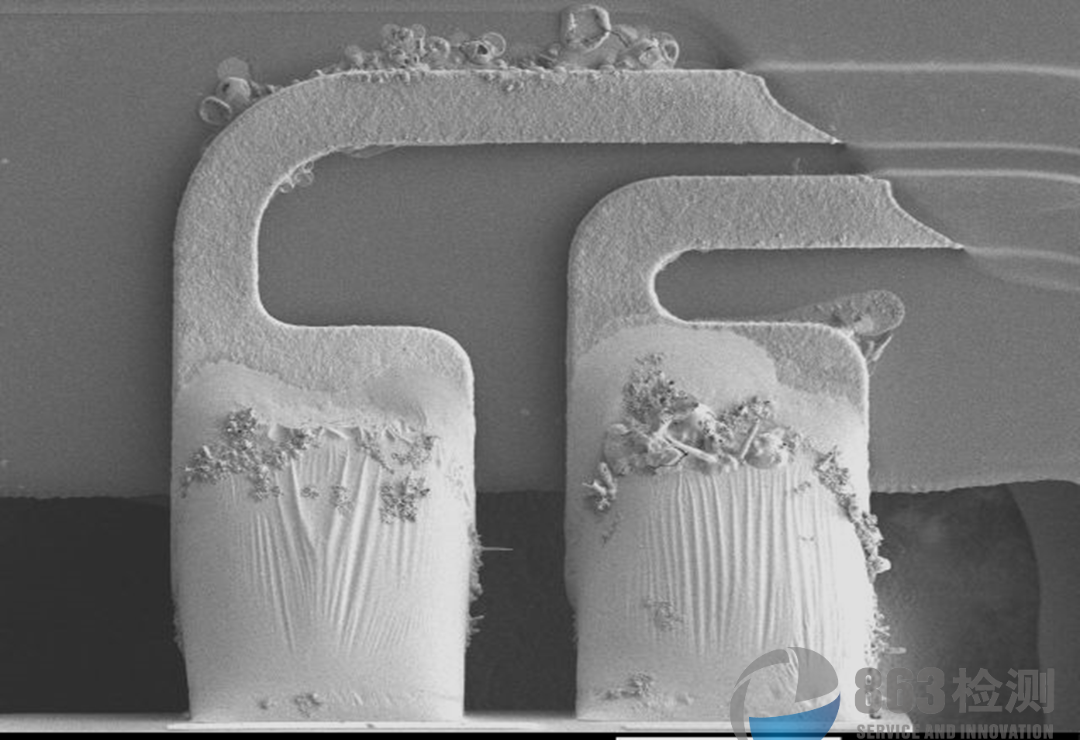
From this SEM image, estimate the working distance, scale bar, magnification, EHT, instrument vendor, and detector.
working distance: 16.5 mm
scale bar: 100000 nm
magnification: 0.25 K X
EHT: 2 kV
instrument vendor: JEOL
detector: LEI